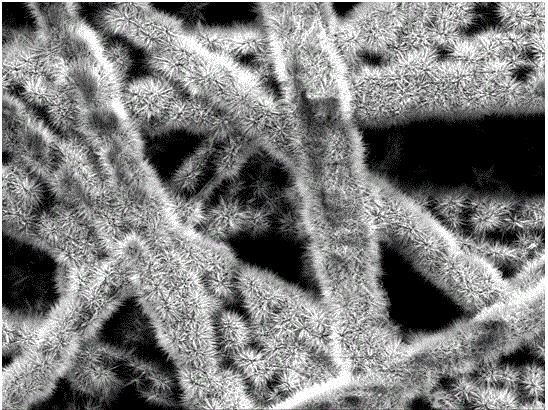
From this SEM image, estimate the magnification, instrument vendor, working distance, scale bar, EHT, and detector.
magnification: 0.5 K X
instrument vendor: JEOL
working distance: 11.5 mm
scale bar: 10000 nm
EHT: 10 kV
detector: SEI